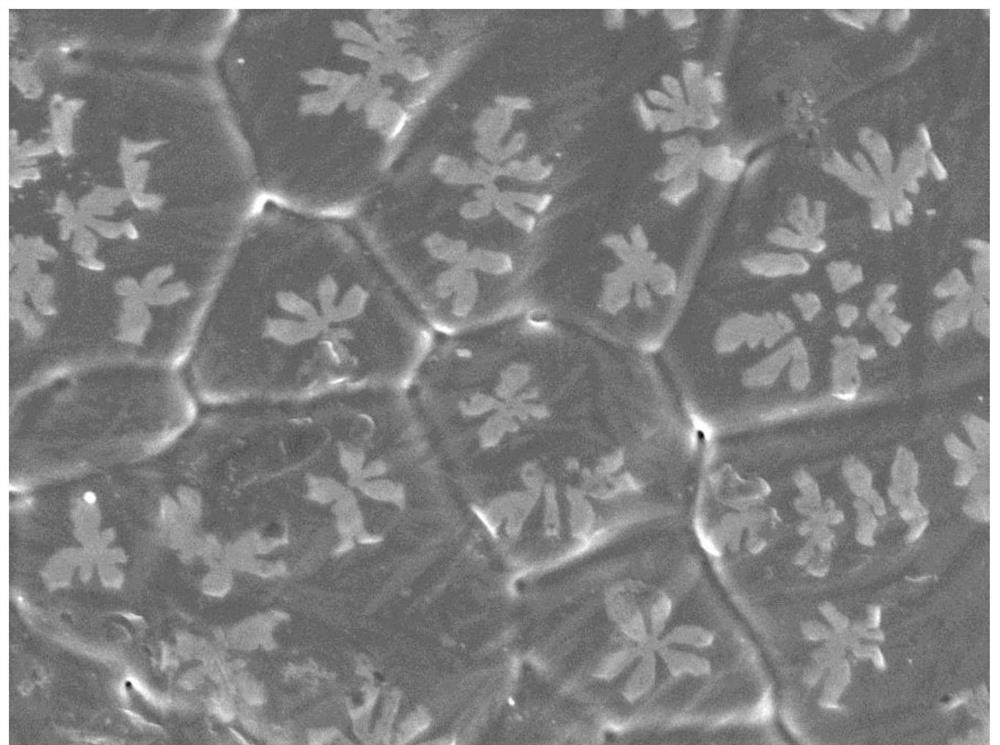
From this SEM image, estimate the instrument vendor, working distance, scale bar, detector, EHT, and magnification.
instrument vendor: JEOL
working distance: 8.8 mm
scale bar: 1000 nm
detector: SEI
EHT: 5 kV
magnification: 10 K X